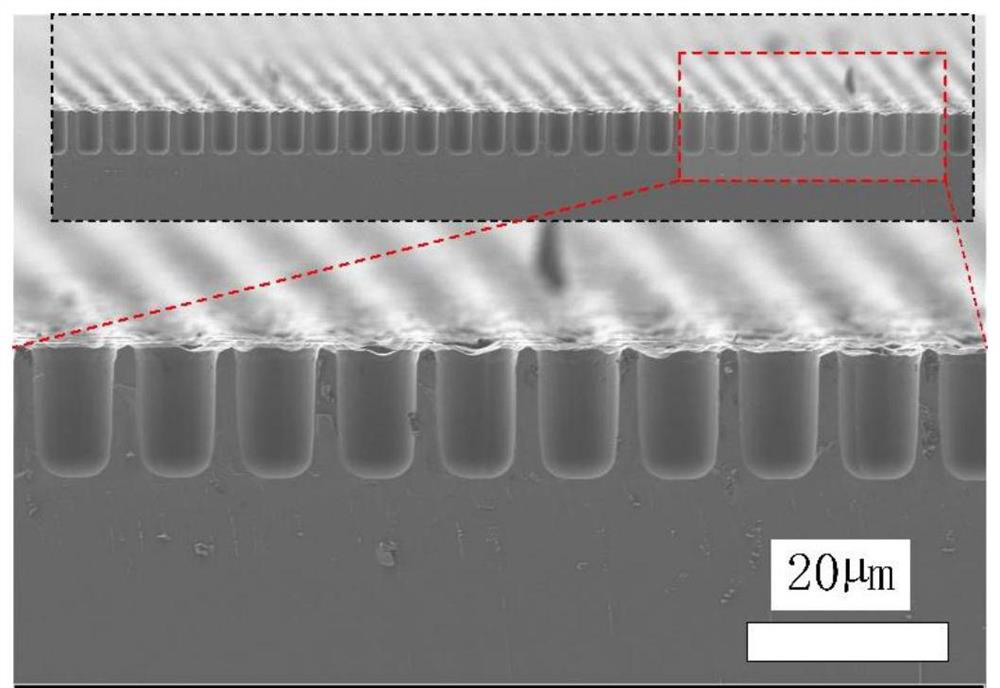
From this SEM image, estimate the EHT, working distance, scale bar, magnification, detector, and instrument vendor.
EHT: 2 kV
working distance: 6.6 mm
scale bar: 1000 nm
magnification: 1.17 K X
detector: SE2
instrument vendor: Zeiss